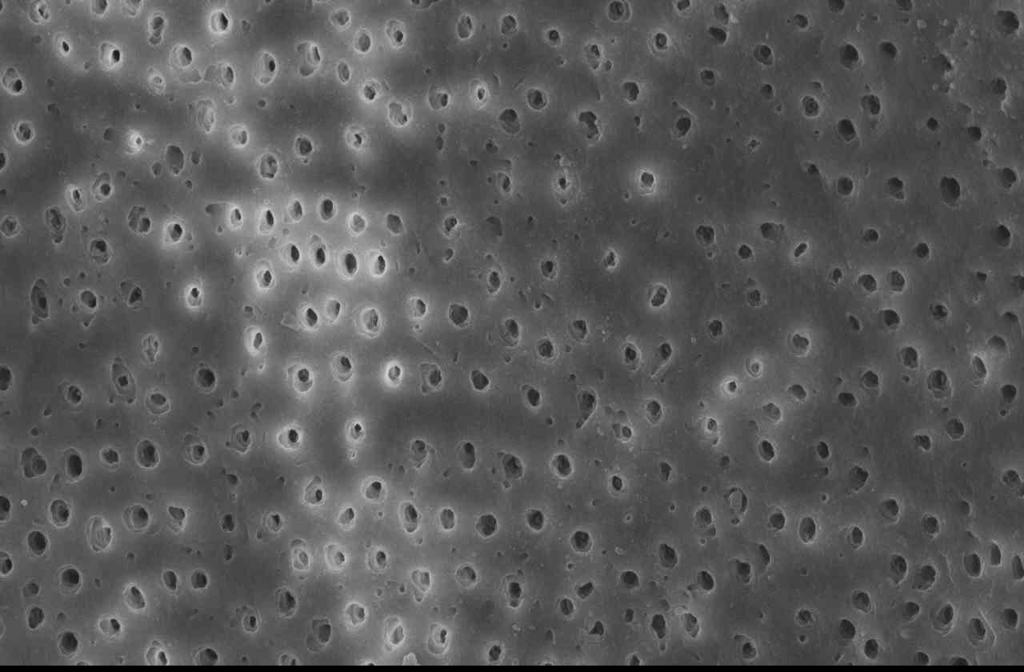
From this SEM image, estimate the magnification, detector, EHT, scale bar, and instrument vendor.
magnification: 1 K X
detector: SEI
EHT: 25 kV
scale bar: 10000 nm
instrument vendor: JEOL